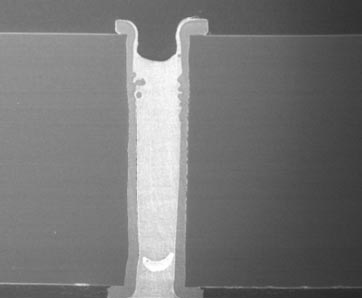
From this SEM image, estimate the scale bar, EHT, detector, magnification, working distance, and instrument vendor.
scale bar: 100000 nm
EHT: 15 kV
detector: SEI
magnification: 0.18 K X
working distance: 22 mm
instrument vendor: JEOL